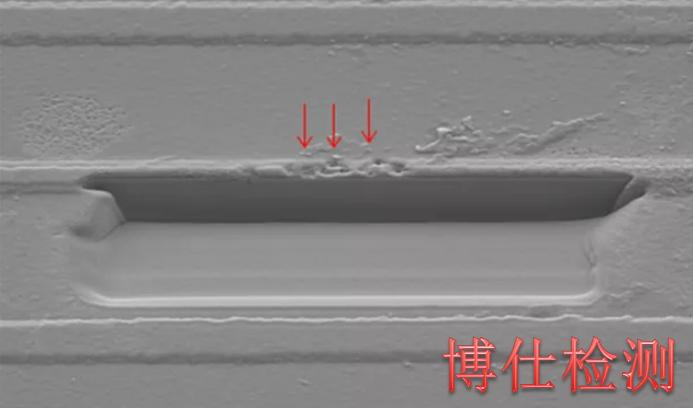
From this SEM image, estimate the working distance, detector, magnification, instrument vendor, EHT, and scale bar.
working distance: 18.1 mm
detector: SE(M)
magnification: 3 K X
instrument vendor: Hitachi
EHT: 5 kV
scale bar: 10000 nm